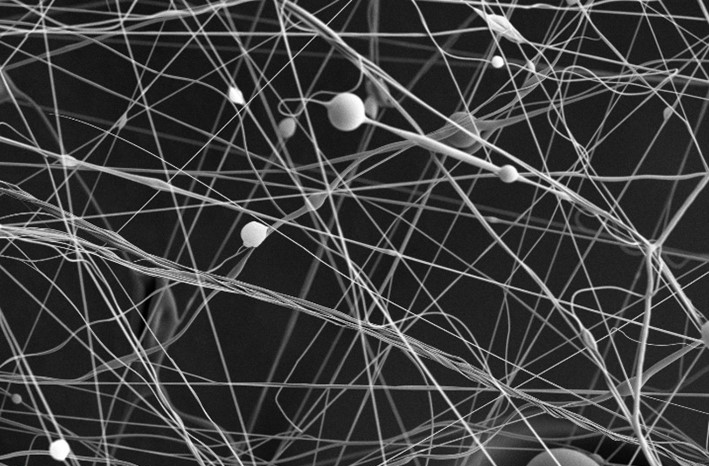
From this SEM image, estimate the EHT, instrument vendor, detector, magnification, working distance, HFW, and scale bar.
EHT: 5 kV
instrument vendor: FEI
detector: ETD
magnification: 0.8 K X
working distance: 11.5 mm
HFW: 259 µm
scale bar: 100000 nm